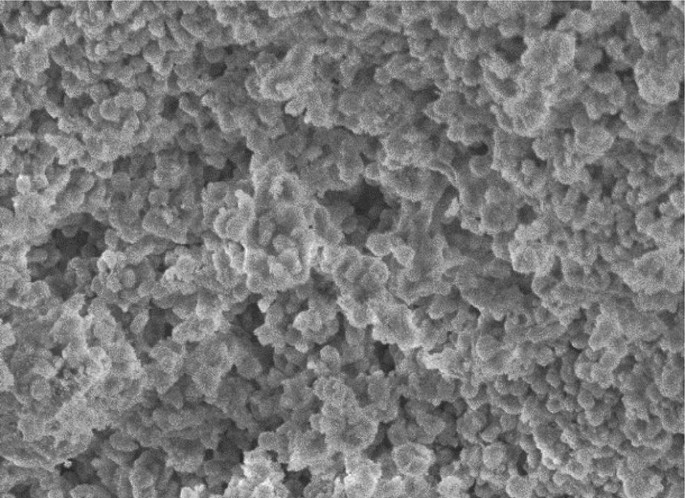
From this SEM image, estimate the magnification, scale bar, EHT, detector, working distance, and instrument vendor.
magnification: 5 K X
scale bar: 10000 nm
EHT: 5 kV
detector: SE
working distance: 14.5 mm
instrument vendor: Hitachi